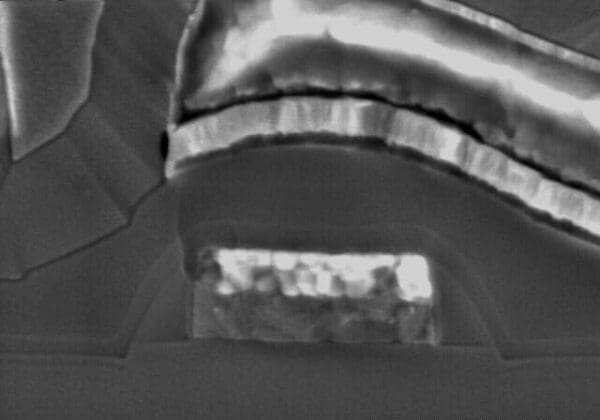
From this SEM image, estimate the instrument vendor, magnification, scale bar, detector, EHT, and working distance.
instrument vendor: Hitachi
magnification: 45 K X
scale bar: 1000 nm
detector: SE(M)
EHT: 5 kV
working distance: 12.3 mm